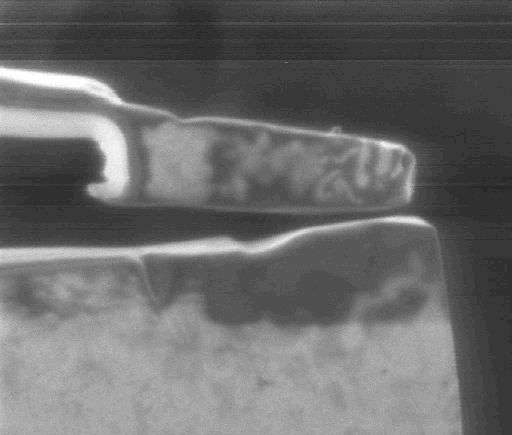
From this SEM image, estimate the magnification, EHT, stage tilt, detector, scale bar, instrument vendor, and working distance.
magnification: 35 K X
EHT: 30 kV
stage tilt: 0°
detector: CDM-E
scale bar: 1000 nm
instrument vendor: FEI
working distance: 17 mm